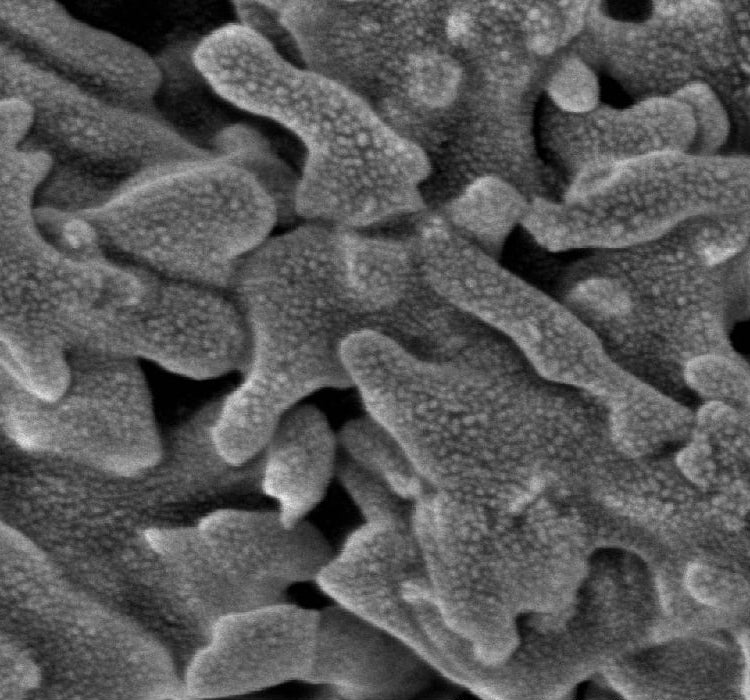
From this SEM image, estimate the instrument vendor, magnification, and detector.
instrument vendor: Hitachi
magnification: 100 K X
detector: SE